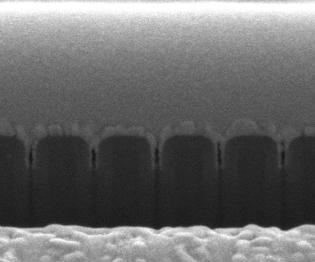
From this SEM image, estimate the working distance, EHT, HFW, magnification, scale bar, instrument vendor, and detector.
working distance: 10 mm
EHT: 5 kV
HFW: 1.49 µm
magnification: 200 K X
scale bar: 500 nm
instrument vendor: FEI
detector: ETD